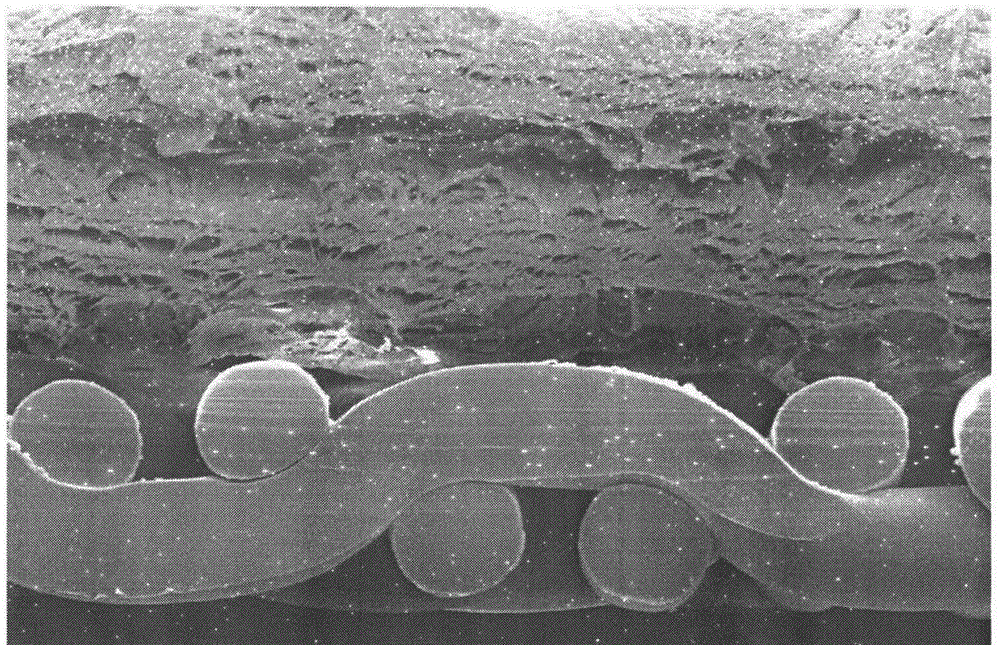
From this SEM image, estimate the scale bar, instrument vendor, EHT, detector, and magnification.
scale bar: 100000 nm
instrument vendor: Hitachi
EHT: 10 kV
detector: SE(M)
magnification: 0.35 K X